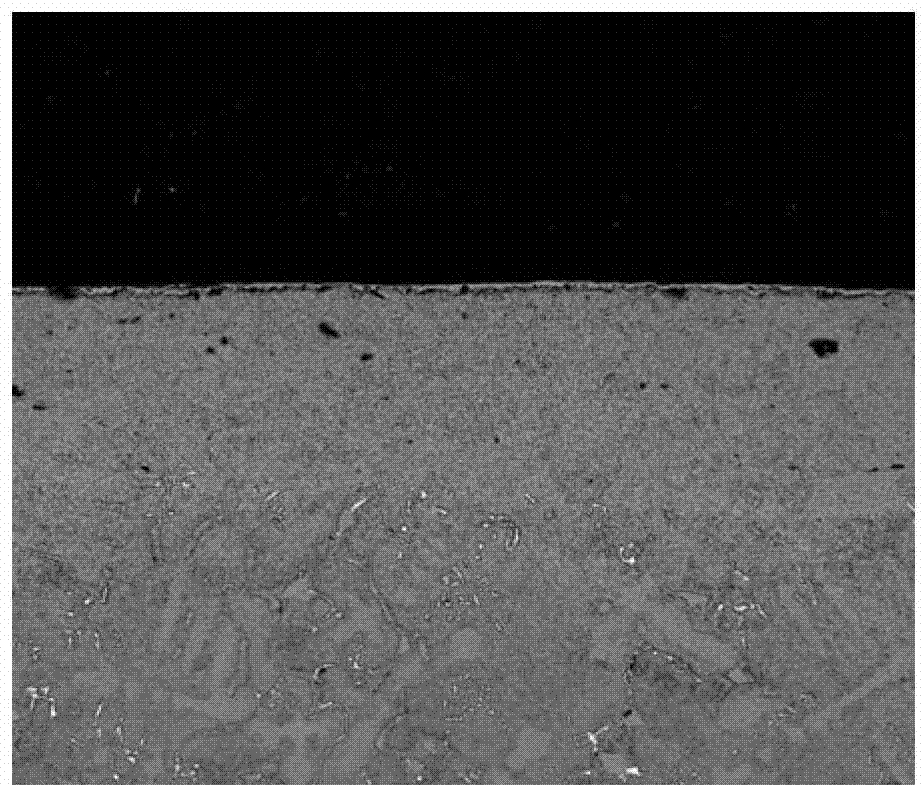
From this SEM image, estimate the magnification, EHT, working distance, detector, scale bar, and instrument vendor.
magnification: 0.25 K X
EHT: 25 kV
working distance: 10.6 mm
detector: A+B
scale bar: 500000 nm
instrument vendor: FEI